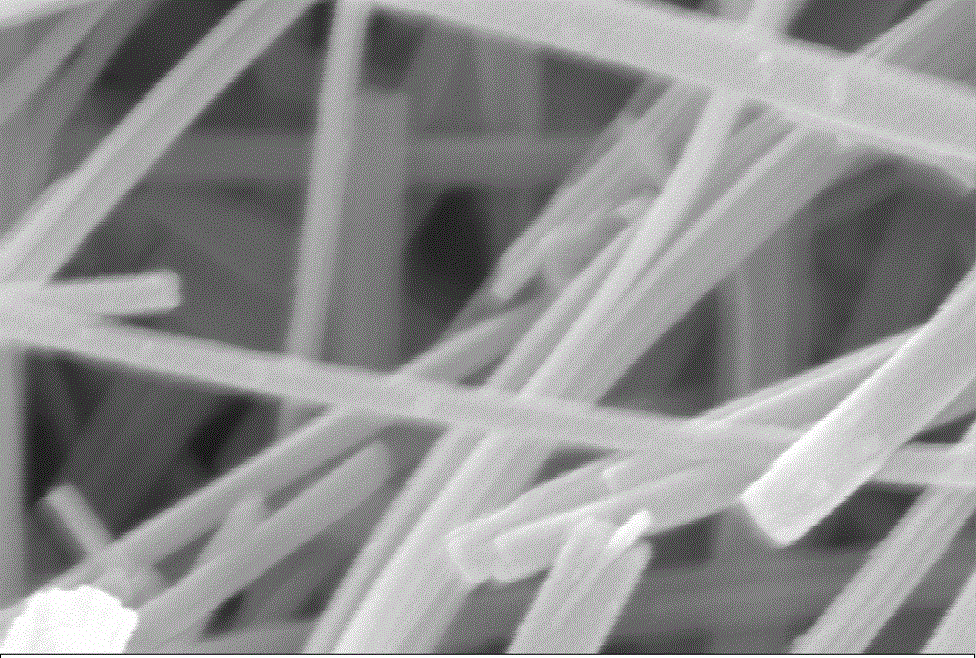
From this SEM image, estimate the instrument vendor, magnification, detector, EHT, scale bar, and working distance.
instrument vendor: Zeiss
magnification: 200 K X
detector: InLens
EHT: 10 kV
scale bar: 100 nm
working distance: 7 mm